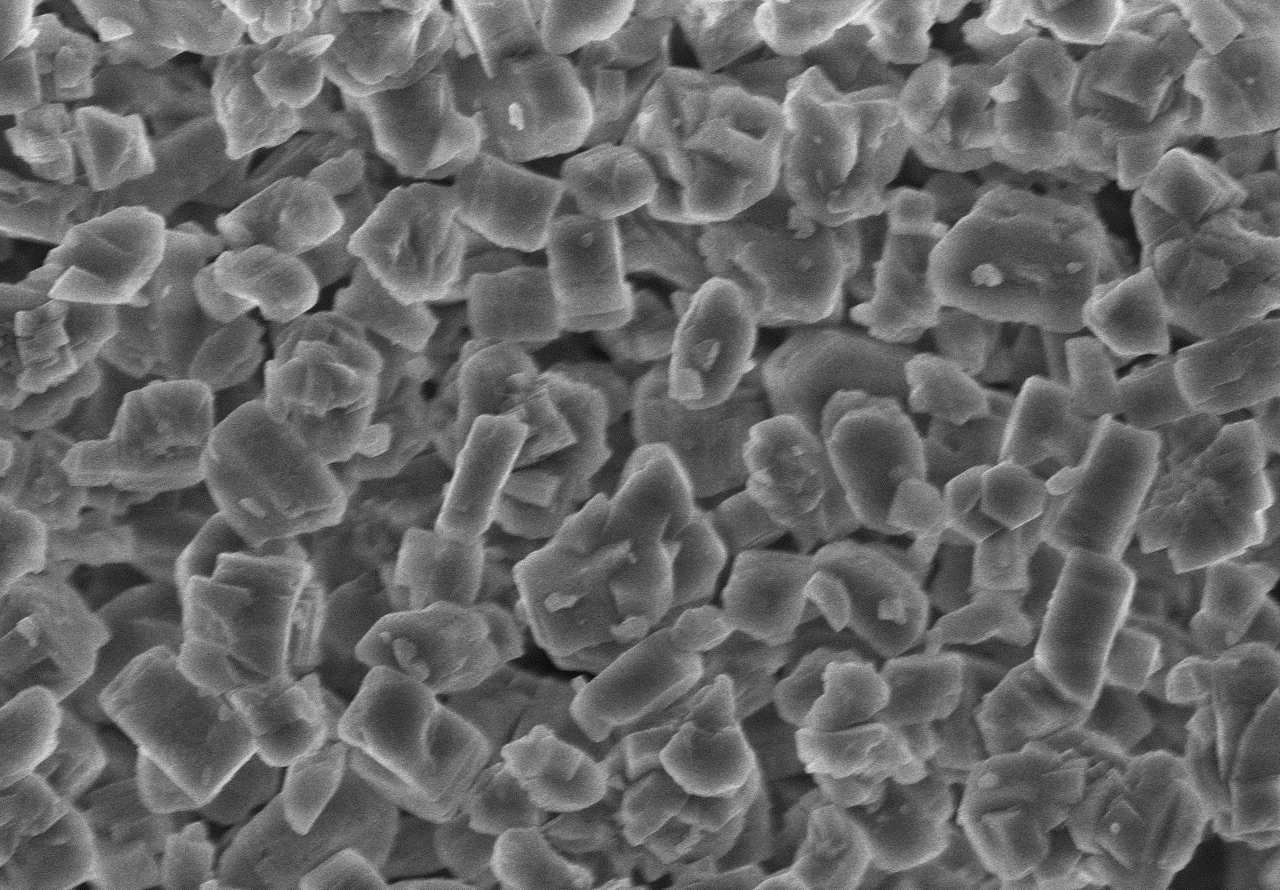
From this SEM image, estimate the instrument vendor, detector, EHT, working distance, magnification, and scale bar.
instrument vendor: Hitachi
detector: SE(M)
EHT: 5 kV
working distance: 3.8 mm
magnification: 8 K X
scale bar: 5000 nm